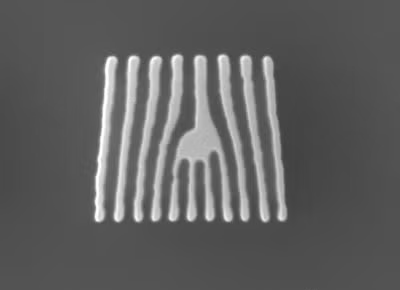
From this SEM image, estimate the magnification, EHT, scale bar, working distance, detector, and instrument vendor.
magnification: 50 K X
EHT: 10 kV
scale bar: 100 nm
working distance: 10.6 mm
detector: LED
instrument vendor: JEOL